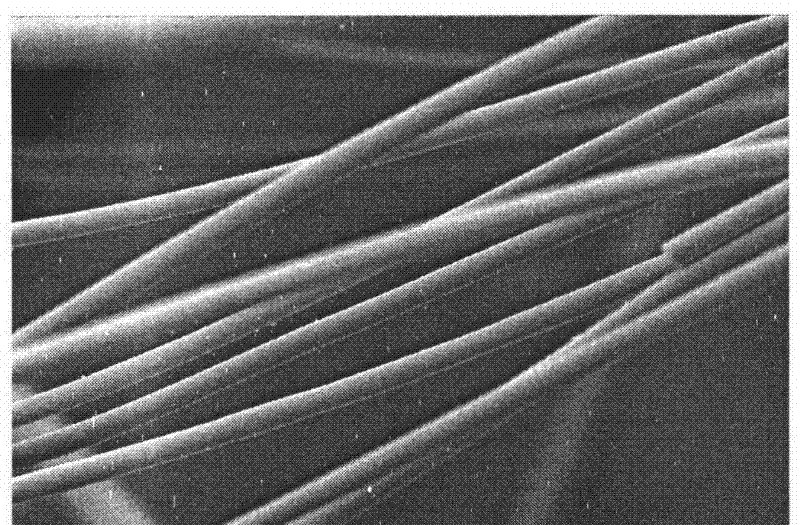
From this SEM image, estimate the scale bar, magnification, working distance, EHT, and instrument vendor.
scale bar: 10000 nm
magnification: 1 K X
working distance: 48 mm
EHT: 20 kV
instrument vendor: JEOL